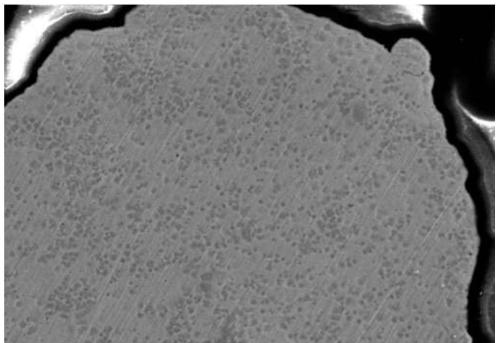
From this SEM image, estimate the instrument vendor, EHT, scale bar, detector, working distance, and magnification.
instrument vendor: Hitachi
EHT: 20 kV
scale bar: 50000 nm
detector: SE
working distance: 9.6 mm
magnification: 1 K X